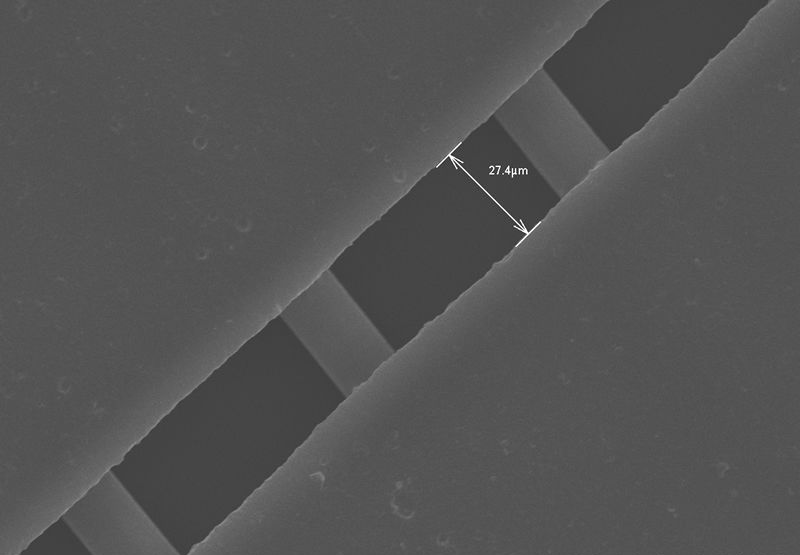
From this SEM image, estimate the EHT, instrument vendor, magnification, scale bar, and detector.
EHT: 30 kV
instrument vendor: Hitachi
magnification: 0.651 K X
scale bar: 50000 nm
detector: SE